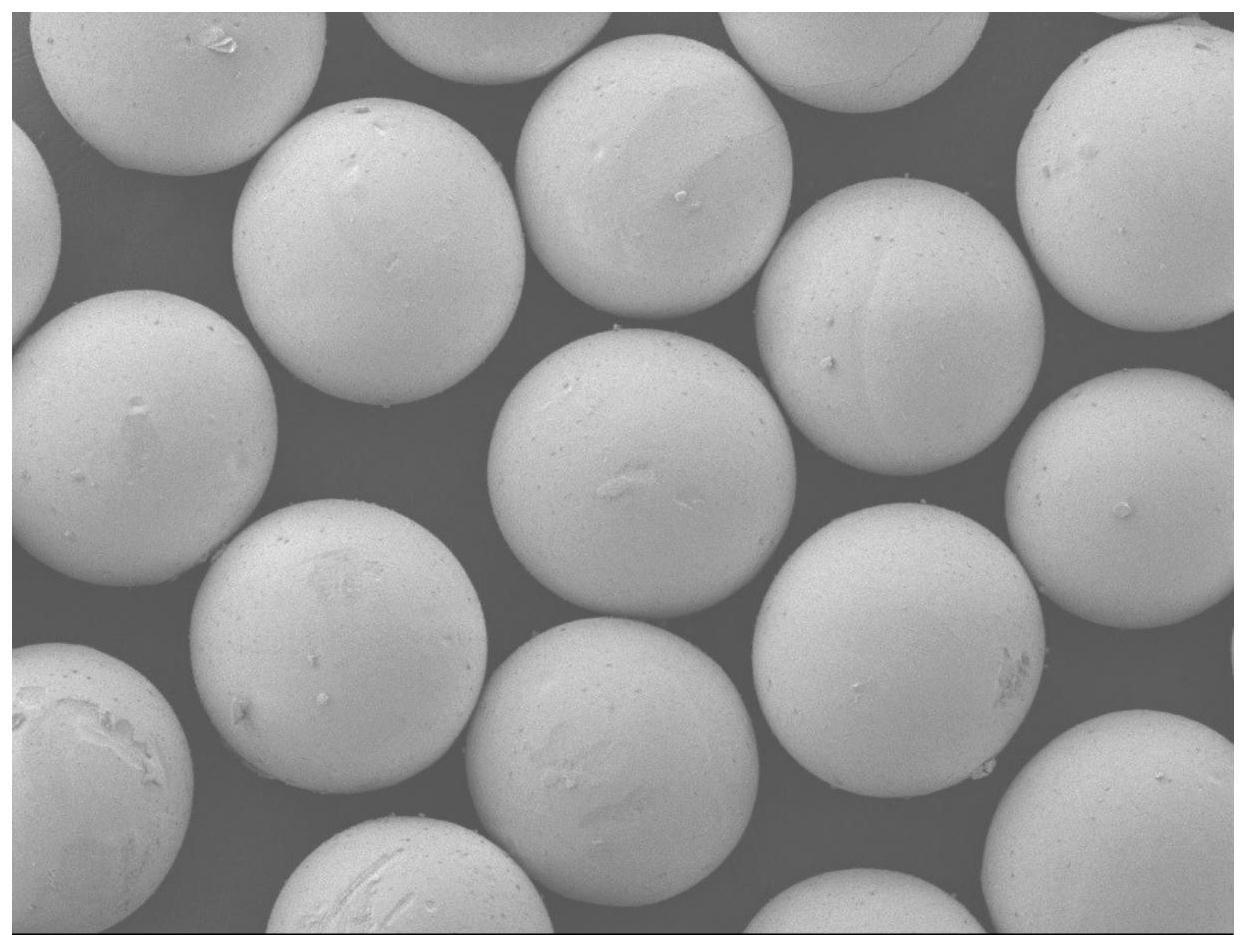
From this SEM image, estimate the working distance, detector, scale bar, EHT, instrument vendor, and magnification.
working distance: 8 mm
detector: LEI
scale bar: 100000 nm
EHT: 3 kV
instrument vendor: JEOL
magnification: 0.07 K X